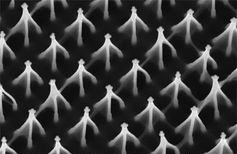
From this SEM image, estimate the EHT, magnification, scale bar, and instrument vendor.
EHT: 20 kV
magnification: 5 K X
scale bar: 5000 nm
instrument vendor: JEOL